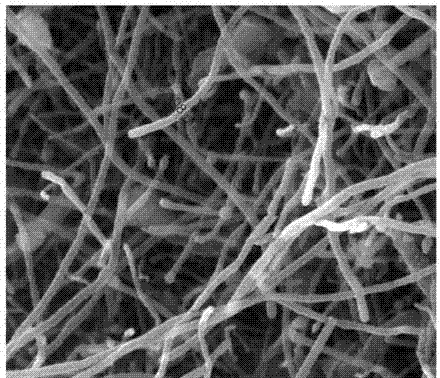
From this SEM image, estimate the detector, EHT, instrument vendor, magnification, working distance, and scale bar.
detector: ETD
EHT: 20 kV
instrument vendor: FEI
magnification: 150 K X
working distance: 11.4 mm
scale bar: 500 nm